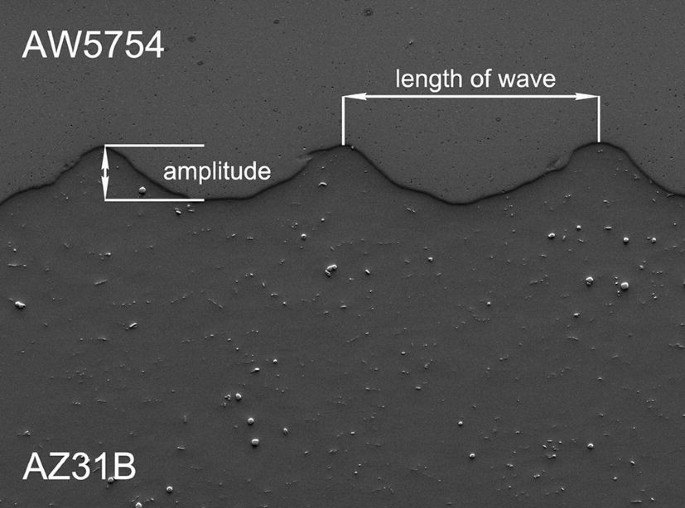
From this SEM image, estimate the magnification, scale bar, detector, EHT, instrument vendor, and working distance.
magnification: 0.033 K X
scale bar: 100000 nm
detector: LEI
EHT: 15 kV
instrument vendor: JEOL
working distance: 34.8 mm